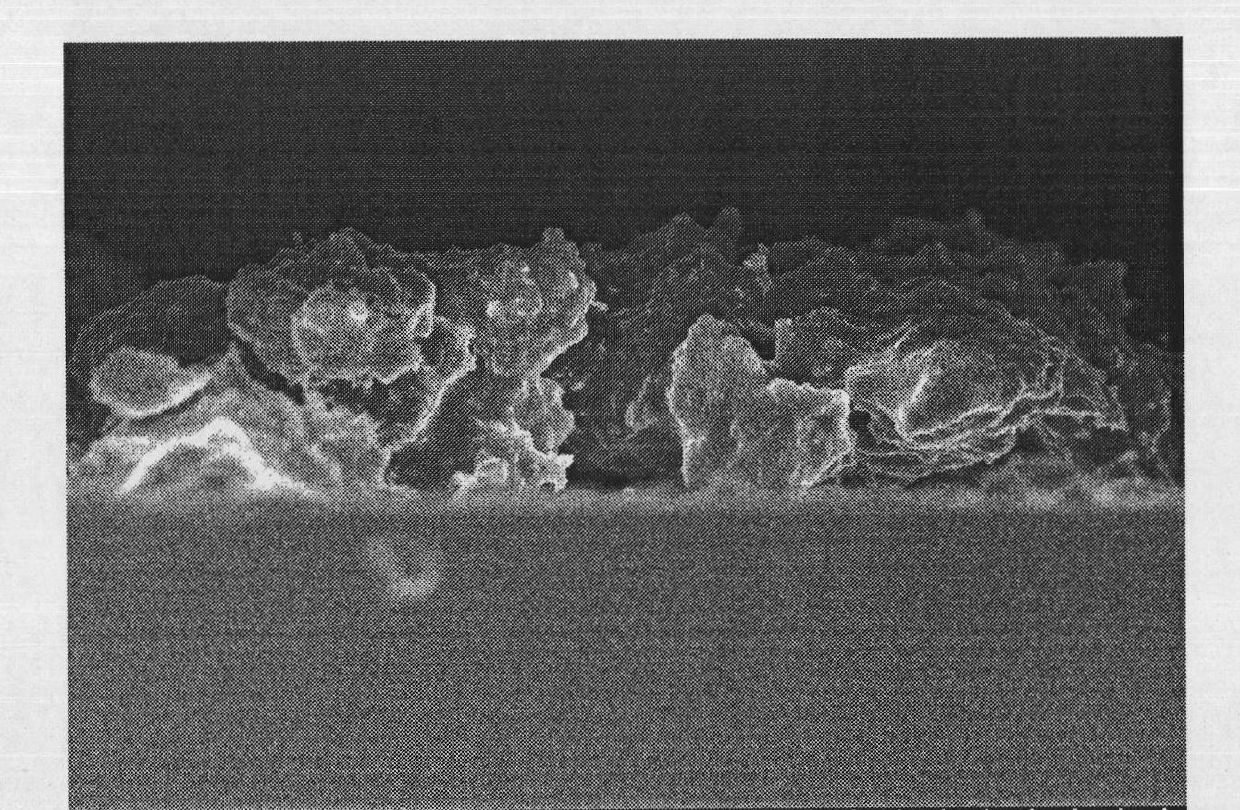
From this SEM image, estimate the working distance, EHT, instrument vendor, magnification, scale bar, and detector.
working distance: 6.5 mm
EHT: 5 kV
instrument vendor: Hitachi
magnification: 5 K X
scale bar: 10000 nm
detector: SE(M)